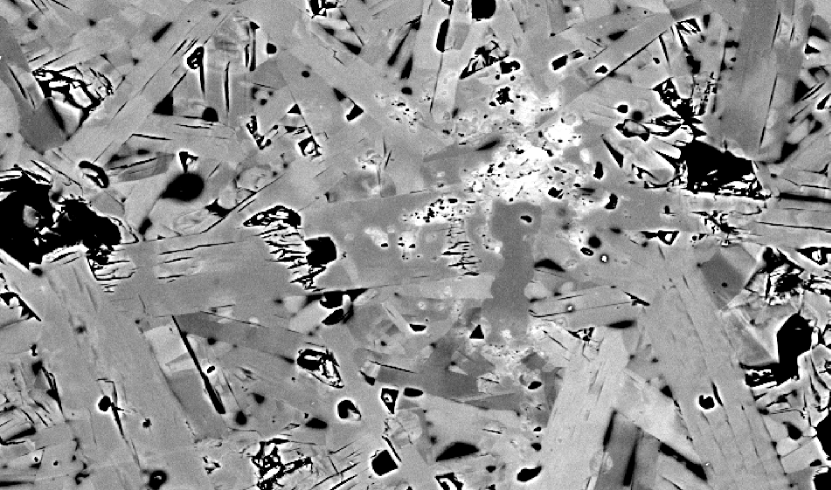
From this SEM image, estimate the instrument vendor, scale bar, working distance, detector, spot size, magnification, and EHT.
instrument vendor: FEI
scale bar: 20000 nm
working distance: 9.6 mm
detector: BSE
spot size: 5.6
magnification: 1 K X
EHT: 20 kV